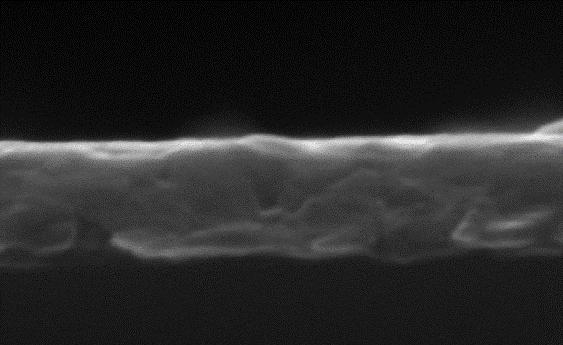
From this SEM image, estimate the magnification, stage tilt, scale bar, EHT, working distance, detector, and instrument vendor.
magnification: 400 K X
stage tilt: -0.2°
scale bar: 100 nm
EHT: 15 kV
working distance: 22.8 mm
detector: SE2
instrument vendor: Zeiss